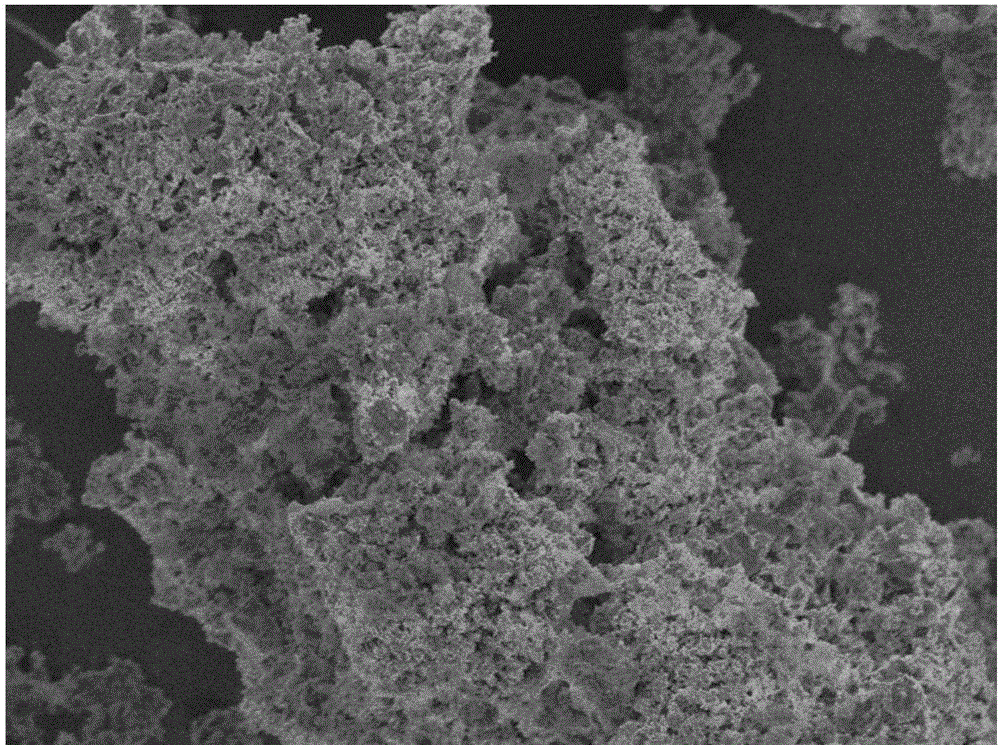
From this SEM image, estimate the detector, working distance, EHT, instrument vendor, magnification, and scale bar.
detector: SEI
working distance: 7 mm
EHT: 5 kV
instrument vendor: JEOL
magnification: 2 K X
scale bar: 10000 nm